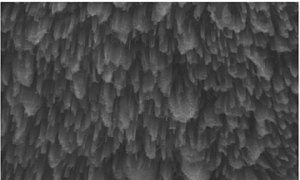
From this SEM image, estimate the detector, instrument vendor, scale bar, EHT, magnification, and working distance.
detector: SE(UL)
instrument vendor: Hitachi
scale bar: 4000 nm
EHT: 15 kV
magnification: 12 K X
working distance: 9 mm